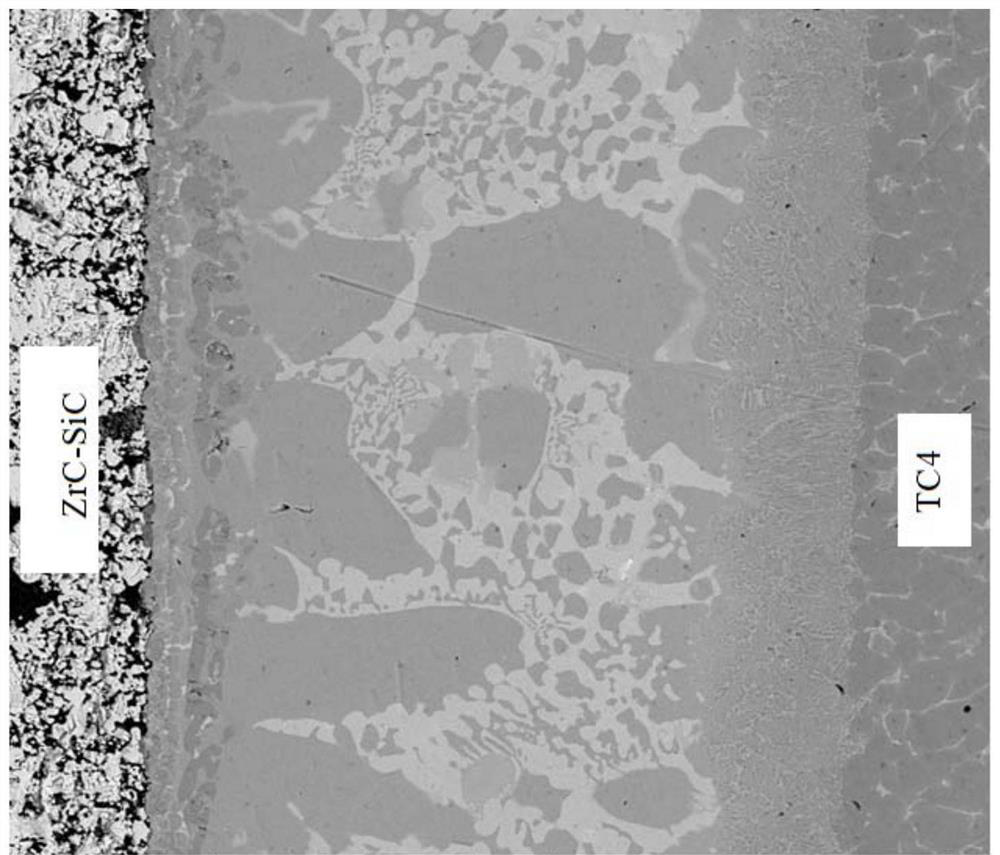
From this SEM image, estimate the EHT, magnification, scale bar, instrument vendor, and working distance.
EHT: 20 kV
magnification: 0.8 K X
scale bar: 100000 nm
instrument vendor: FEI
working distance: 11.5 mm